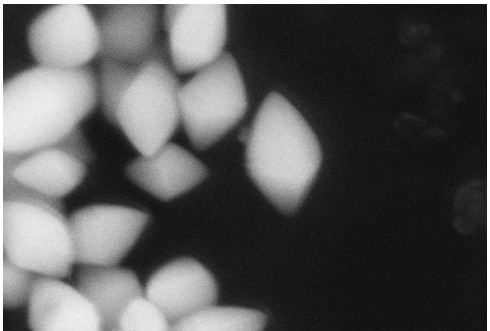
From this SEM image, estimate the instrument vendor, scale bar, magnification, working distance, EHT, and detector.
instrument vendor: Zeiss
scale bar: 200 nm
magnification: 80 K X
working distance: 9.4 mm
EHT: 10 kV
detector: SE2